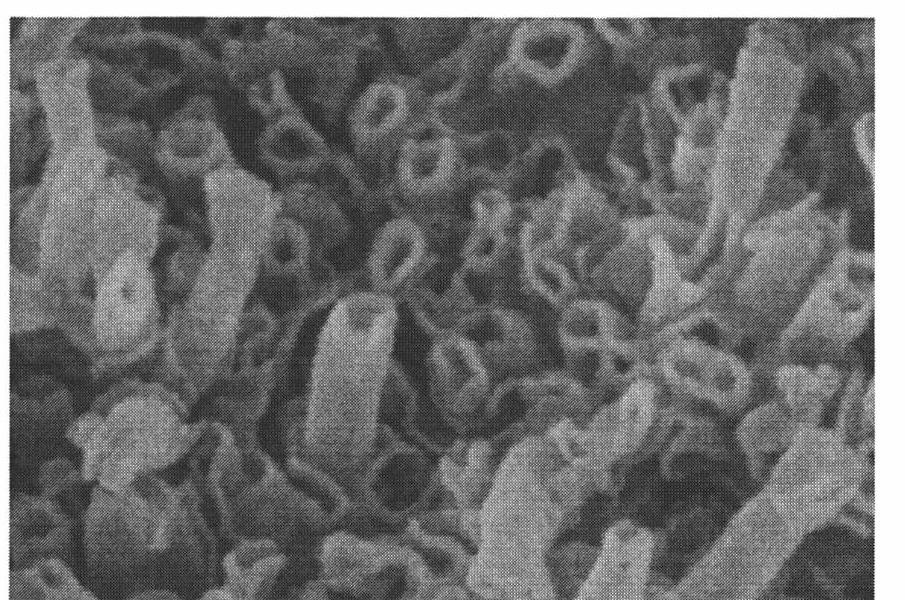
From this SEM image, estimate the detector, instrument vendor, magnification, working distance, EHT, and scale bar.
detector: SE(U)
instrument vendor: Hitachi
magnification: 200 K X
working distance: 5.1 mm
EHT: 5 kV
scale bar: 200 nm